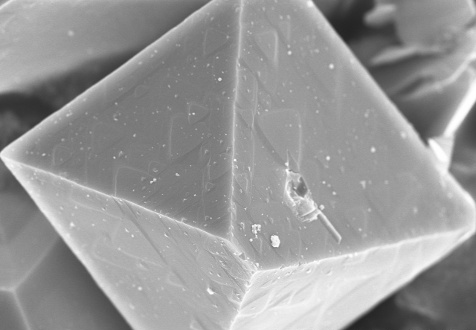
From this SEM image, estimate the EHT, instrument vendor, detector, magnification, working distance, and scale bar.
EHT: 10 kV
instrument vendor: Hitachi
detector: SE(U)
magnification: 5 K X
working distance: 4.2 mm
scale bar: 10000 nm